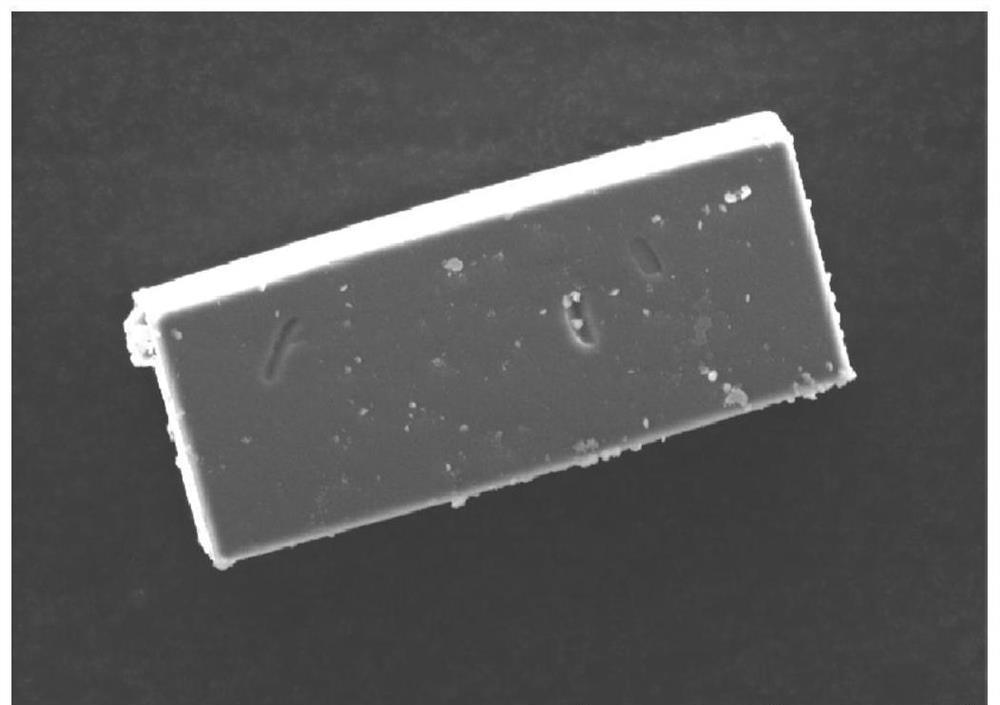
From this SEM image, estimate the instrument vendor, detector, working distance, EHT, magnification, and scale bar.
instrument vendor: Hitachi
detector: LM(L)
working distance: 9.3 mm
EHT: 15 kV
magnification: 1 K X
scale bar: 50000 nm